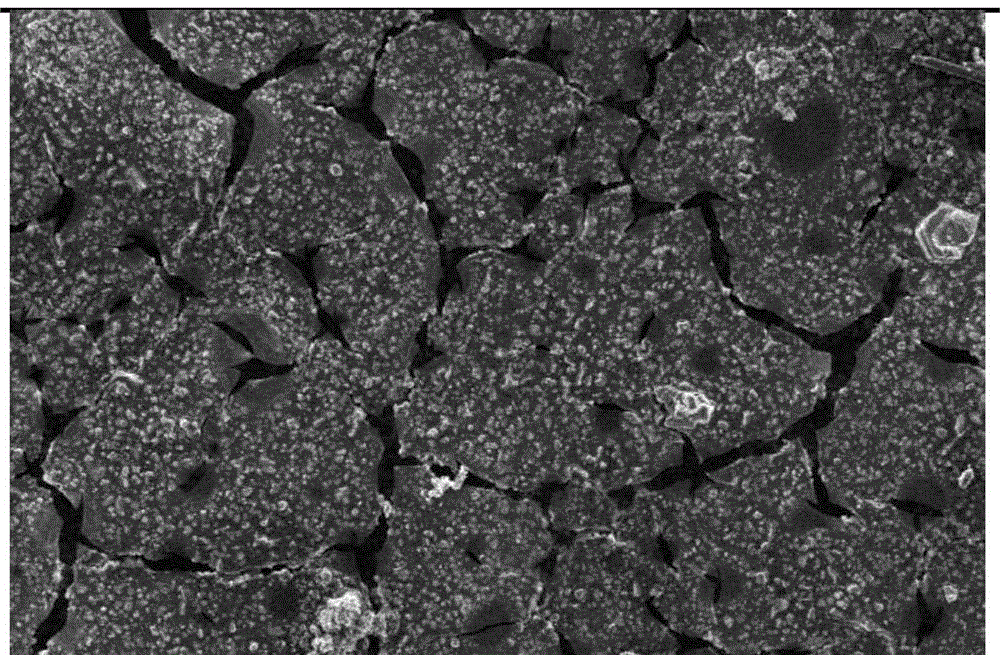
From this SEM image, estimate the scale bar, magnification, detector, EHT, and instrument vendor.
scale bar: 100000 nm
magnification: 0.1 K X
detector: SEI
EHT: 15 kV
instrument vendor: JEOL